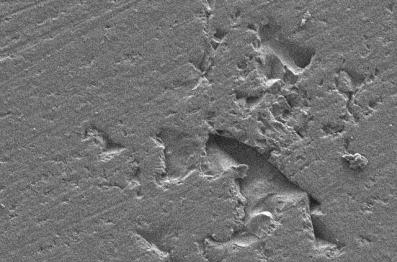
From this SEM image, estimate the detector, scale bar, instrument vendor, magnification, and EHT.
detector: SEI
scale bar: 10000 nm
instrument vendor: JEOL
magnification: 1 K X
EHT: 10 kV